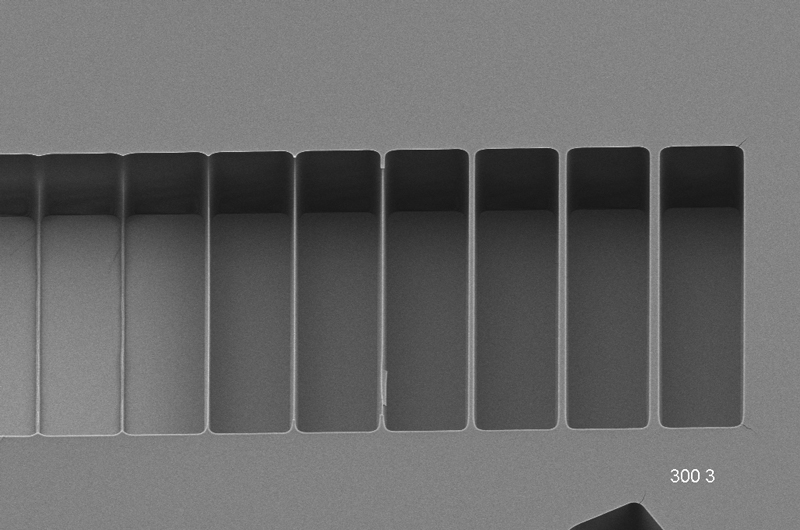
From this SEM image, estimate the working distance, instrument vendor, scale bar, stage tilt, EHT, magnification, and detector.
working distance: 6.7 mm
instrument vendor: Zeiss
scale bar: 10000 nm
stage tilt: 45°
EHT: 3 kV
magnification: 1.12 K X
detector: HE-SE2